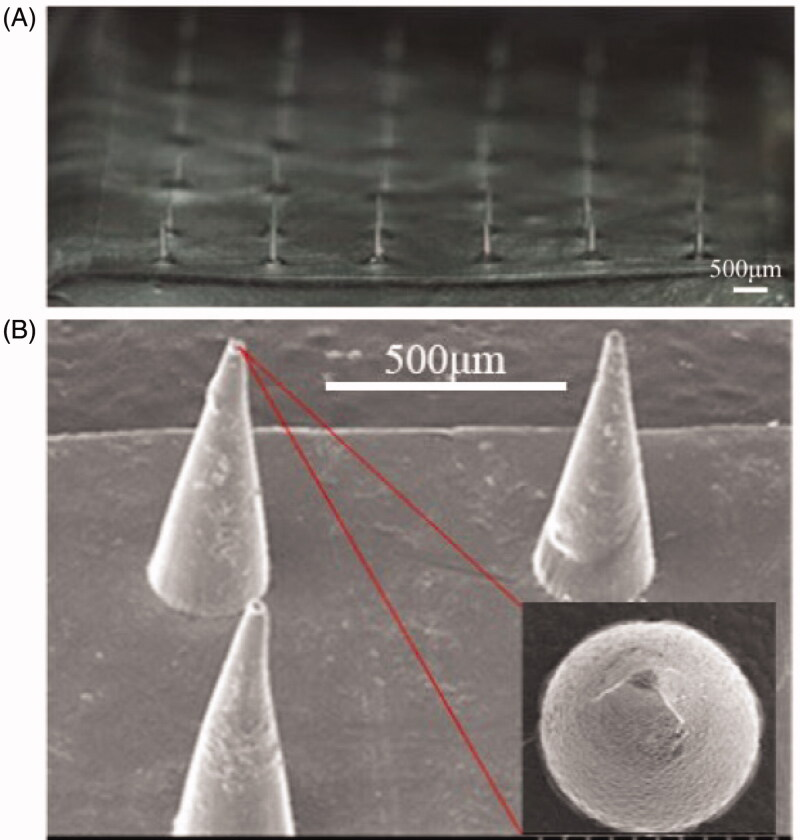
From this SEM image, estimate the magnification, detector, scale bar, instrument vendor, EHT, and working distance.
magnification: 0.08 K X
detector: SE(U)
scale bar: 500000 nm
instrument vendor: Hitachi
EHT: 15 kV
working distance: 10.8 mm